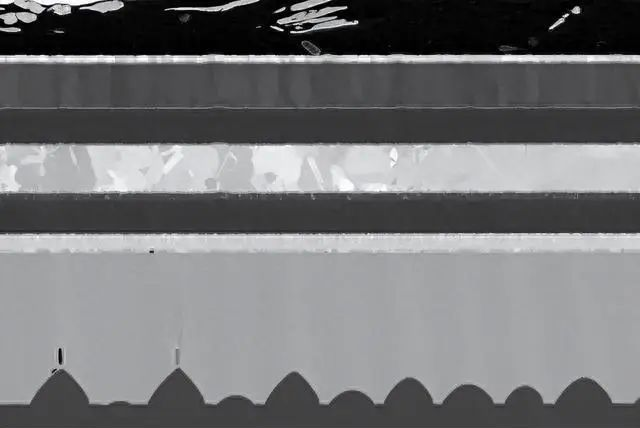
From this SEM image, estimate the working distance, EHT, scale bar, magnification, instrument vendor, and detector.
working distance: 4.9 mm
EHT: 2 kV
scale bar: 1000 nm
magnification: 4 K X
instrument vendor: Zeiss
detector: SE2